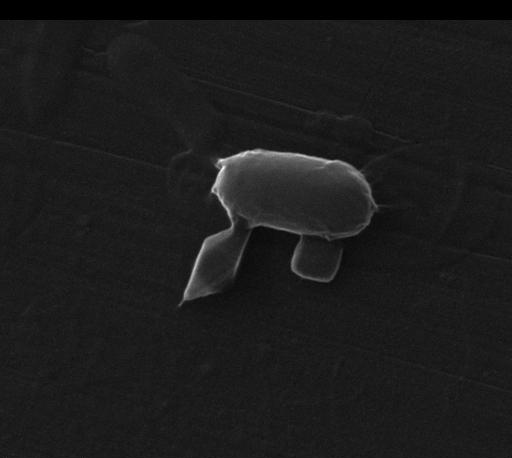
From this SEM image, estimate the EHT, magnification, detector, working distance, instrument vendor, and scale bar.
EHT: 30 kV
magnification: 50 K X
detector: ETD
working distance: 10 mm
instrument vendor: FEI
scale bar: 2000 nm